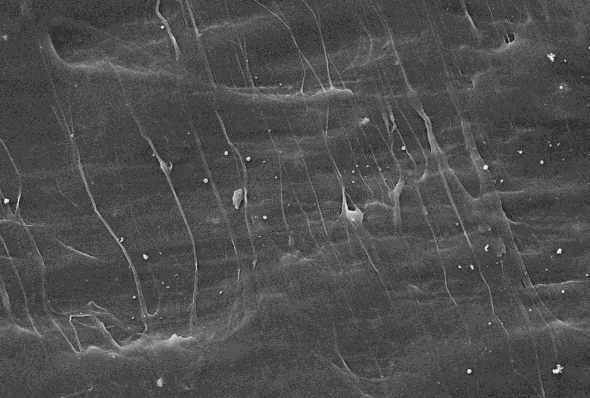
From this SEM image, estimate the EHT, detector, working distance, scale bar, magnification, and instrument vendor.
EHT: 10 kV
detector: SE2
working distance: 16 mm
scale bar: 10000 nm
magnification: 5 K X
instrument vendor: Zeiss